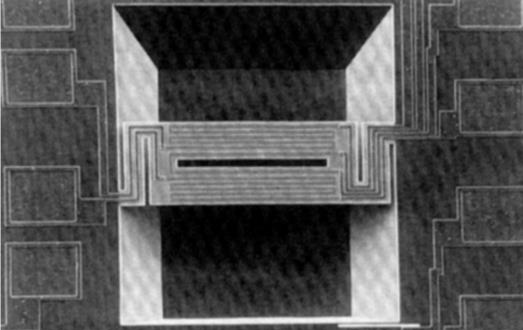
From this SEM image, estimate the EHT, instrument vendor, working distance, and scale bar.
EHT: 15 kV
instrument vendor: JEOL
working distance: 39 mm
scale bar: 100000 nm